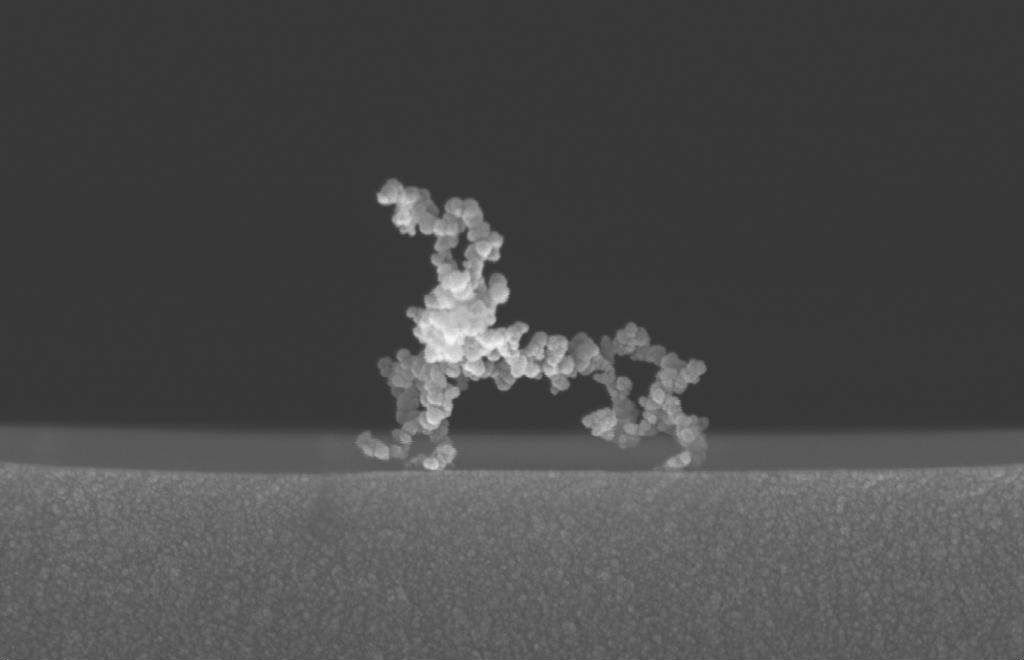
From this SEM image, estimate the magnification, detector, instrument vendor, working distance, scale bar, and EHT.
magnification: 122.14 K X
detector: InLens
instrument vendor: Zeiss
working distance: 6 mm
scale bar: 200 nm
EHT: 5 kV